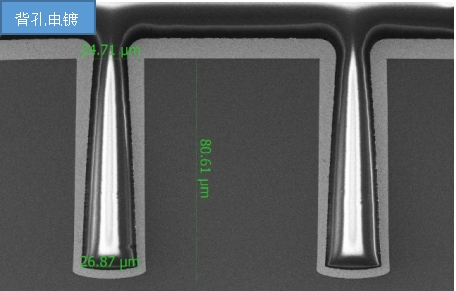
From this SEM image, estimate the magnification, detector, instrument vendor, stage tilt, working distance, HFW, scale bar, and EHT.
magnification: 2.5 K X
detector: ETD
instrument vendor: FEI/Thermo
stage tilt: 0°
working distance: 10.5615 mm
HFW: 166 µm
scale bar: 40000 nm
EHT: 5 kV